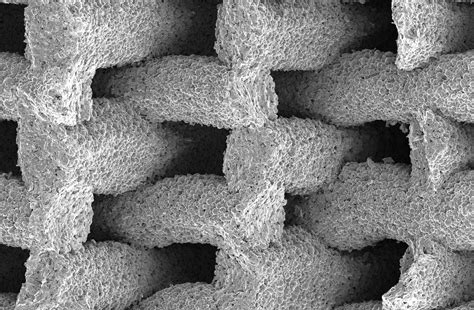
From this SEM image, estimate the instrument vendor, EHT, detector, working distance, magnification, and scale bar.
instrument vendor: FEI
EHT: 5 kV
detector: ETD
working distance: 7.6 mm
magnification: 0.12 K X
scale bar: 500000 nm